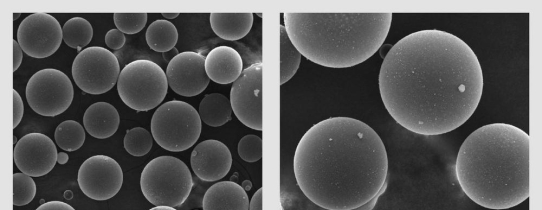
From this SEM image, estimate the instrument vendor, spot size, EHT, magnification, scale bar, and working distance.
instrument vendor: FEI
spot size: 3.5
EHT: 25 kV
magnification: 0.5 K X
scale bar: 200000 nm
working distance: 10.2 mm